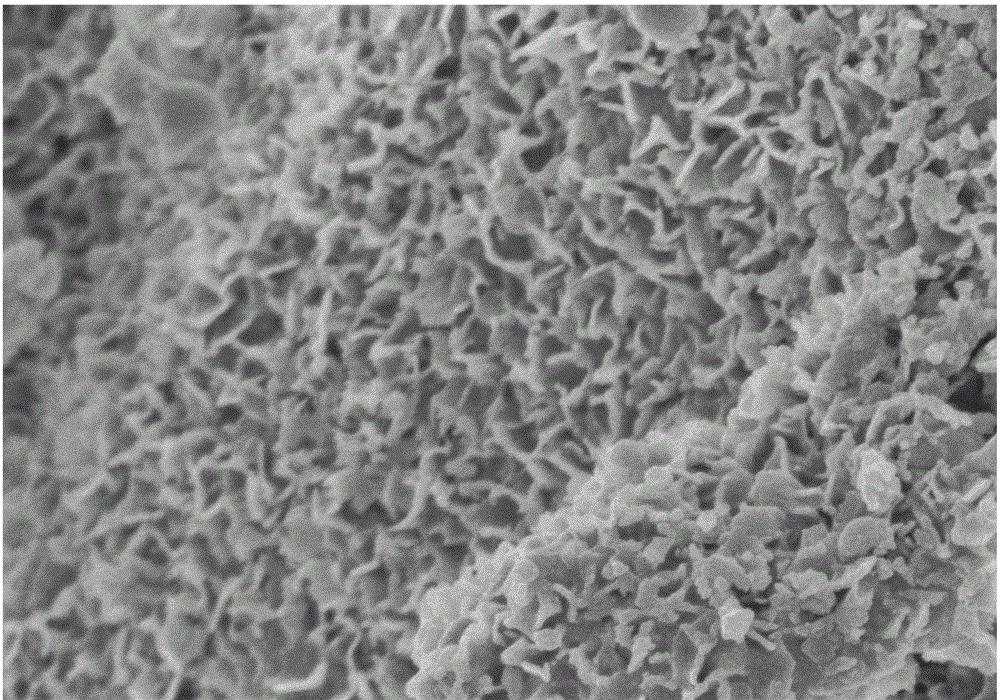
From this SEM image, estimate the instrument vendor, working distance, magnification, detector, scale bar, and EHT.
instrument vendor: Hitachi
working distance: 9.7 mm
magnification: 40 K X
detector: SE(U)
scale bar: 1000 nm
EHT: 3 kV